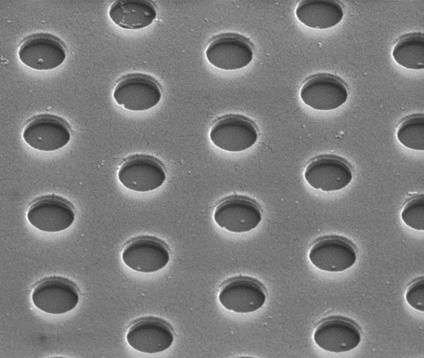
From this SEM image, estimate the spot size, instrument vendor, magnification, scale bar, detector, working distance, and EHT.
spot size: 5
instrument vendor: FEI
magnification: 30 K X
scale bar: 1000 nm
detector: ETD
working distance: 9.4 mm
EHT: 10 kV